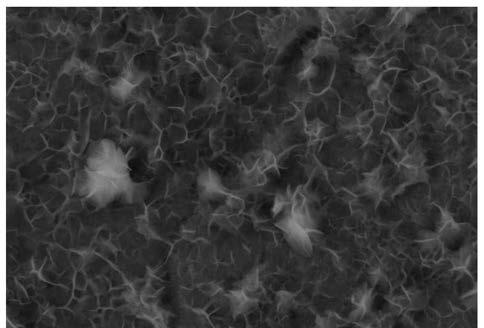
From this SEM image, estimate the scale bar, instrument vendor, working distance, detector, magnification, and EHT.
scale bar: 1000 nm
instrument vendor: Zeiss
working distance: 5.7 mm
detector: InLens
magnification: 10 K X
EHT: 10 kV